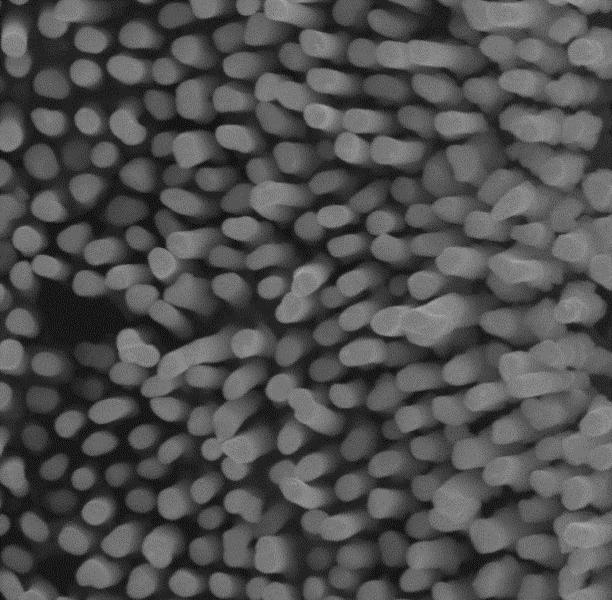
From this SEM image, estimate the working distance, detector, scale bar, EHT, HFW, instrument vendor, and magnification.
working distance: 2.754 mm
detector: InBeam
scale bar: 500 nm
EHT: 15 kV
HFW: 2.167 µm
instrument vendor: Tescan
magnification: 100 K X